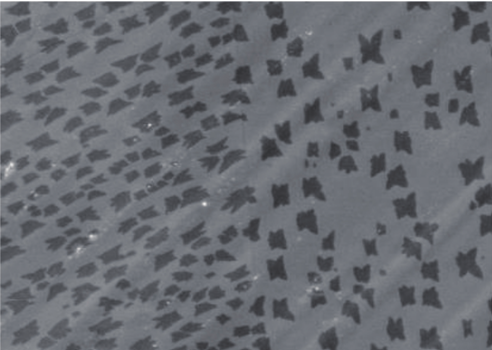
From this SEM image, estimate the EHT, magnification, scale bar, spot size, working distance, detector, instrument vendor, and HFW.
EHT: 30 kV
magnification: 1.6 K X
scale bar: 50000 nm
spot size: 6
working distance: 10.5 mm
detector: ETD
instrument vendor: FEI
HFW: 186 µm